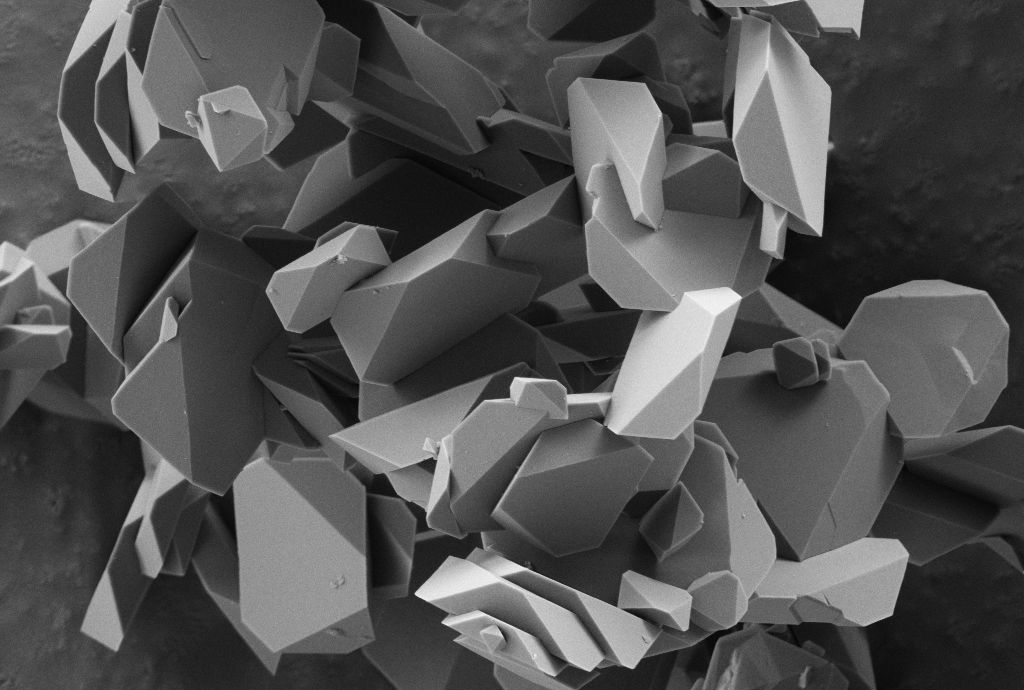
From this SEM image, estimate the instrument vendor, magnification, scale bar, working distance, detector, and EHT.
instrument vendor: Zeiss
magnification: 5 K X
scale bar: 1000 nm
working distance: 3.1 mm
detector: SE2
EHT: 3 kV